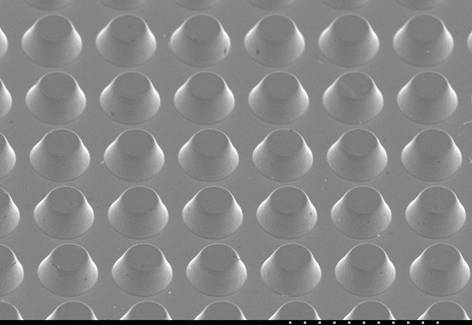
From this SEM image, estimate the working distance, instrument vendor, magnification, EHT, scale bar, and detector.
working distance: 10.7 mm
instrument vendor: Hitachi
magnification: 2 K X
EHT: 5 kV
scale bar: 20000 nm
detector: SE